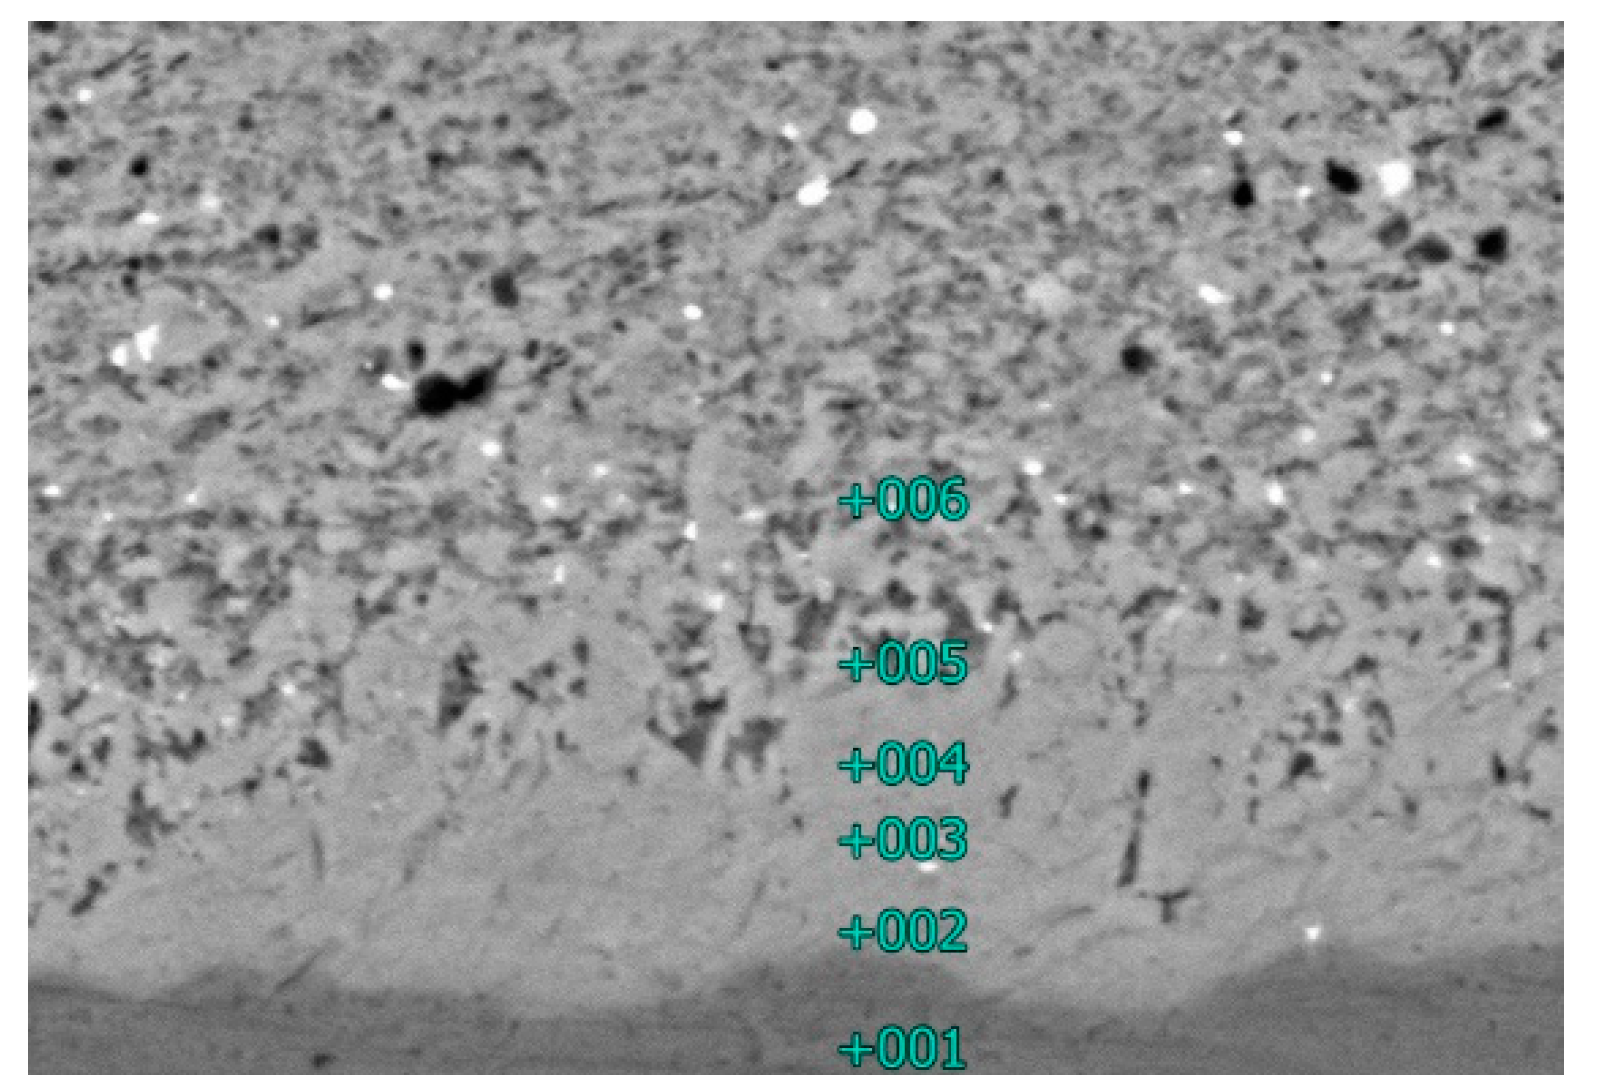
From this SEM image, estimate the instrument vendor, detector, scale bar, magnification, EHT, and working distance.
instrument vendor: JEOL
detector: BEC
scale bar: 2000 nm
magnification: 7 K X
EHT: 15 kV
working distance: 10 mm